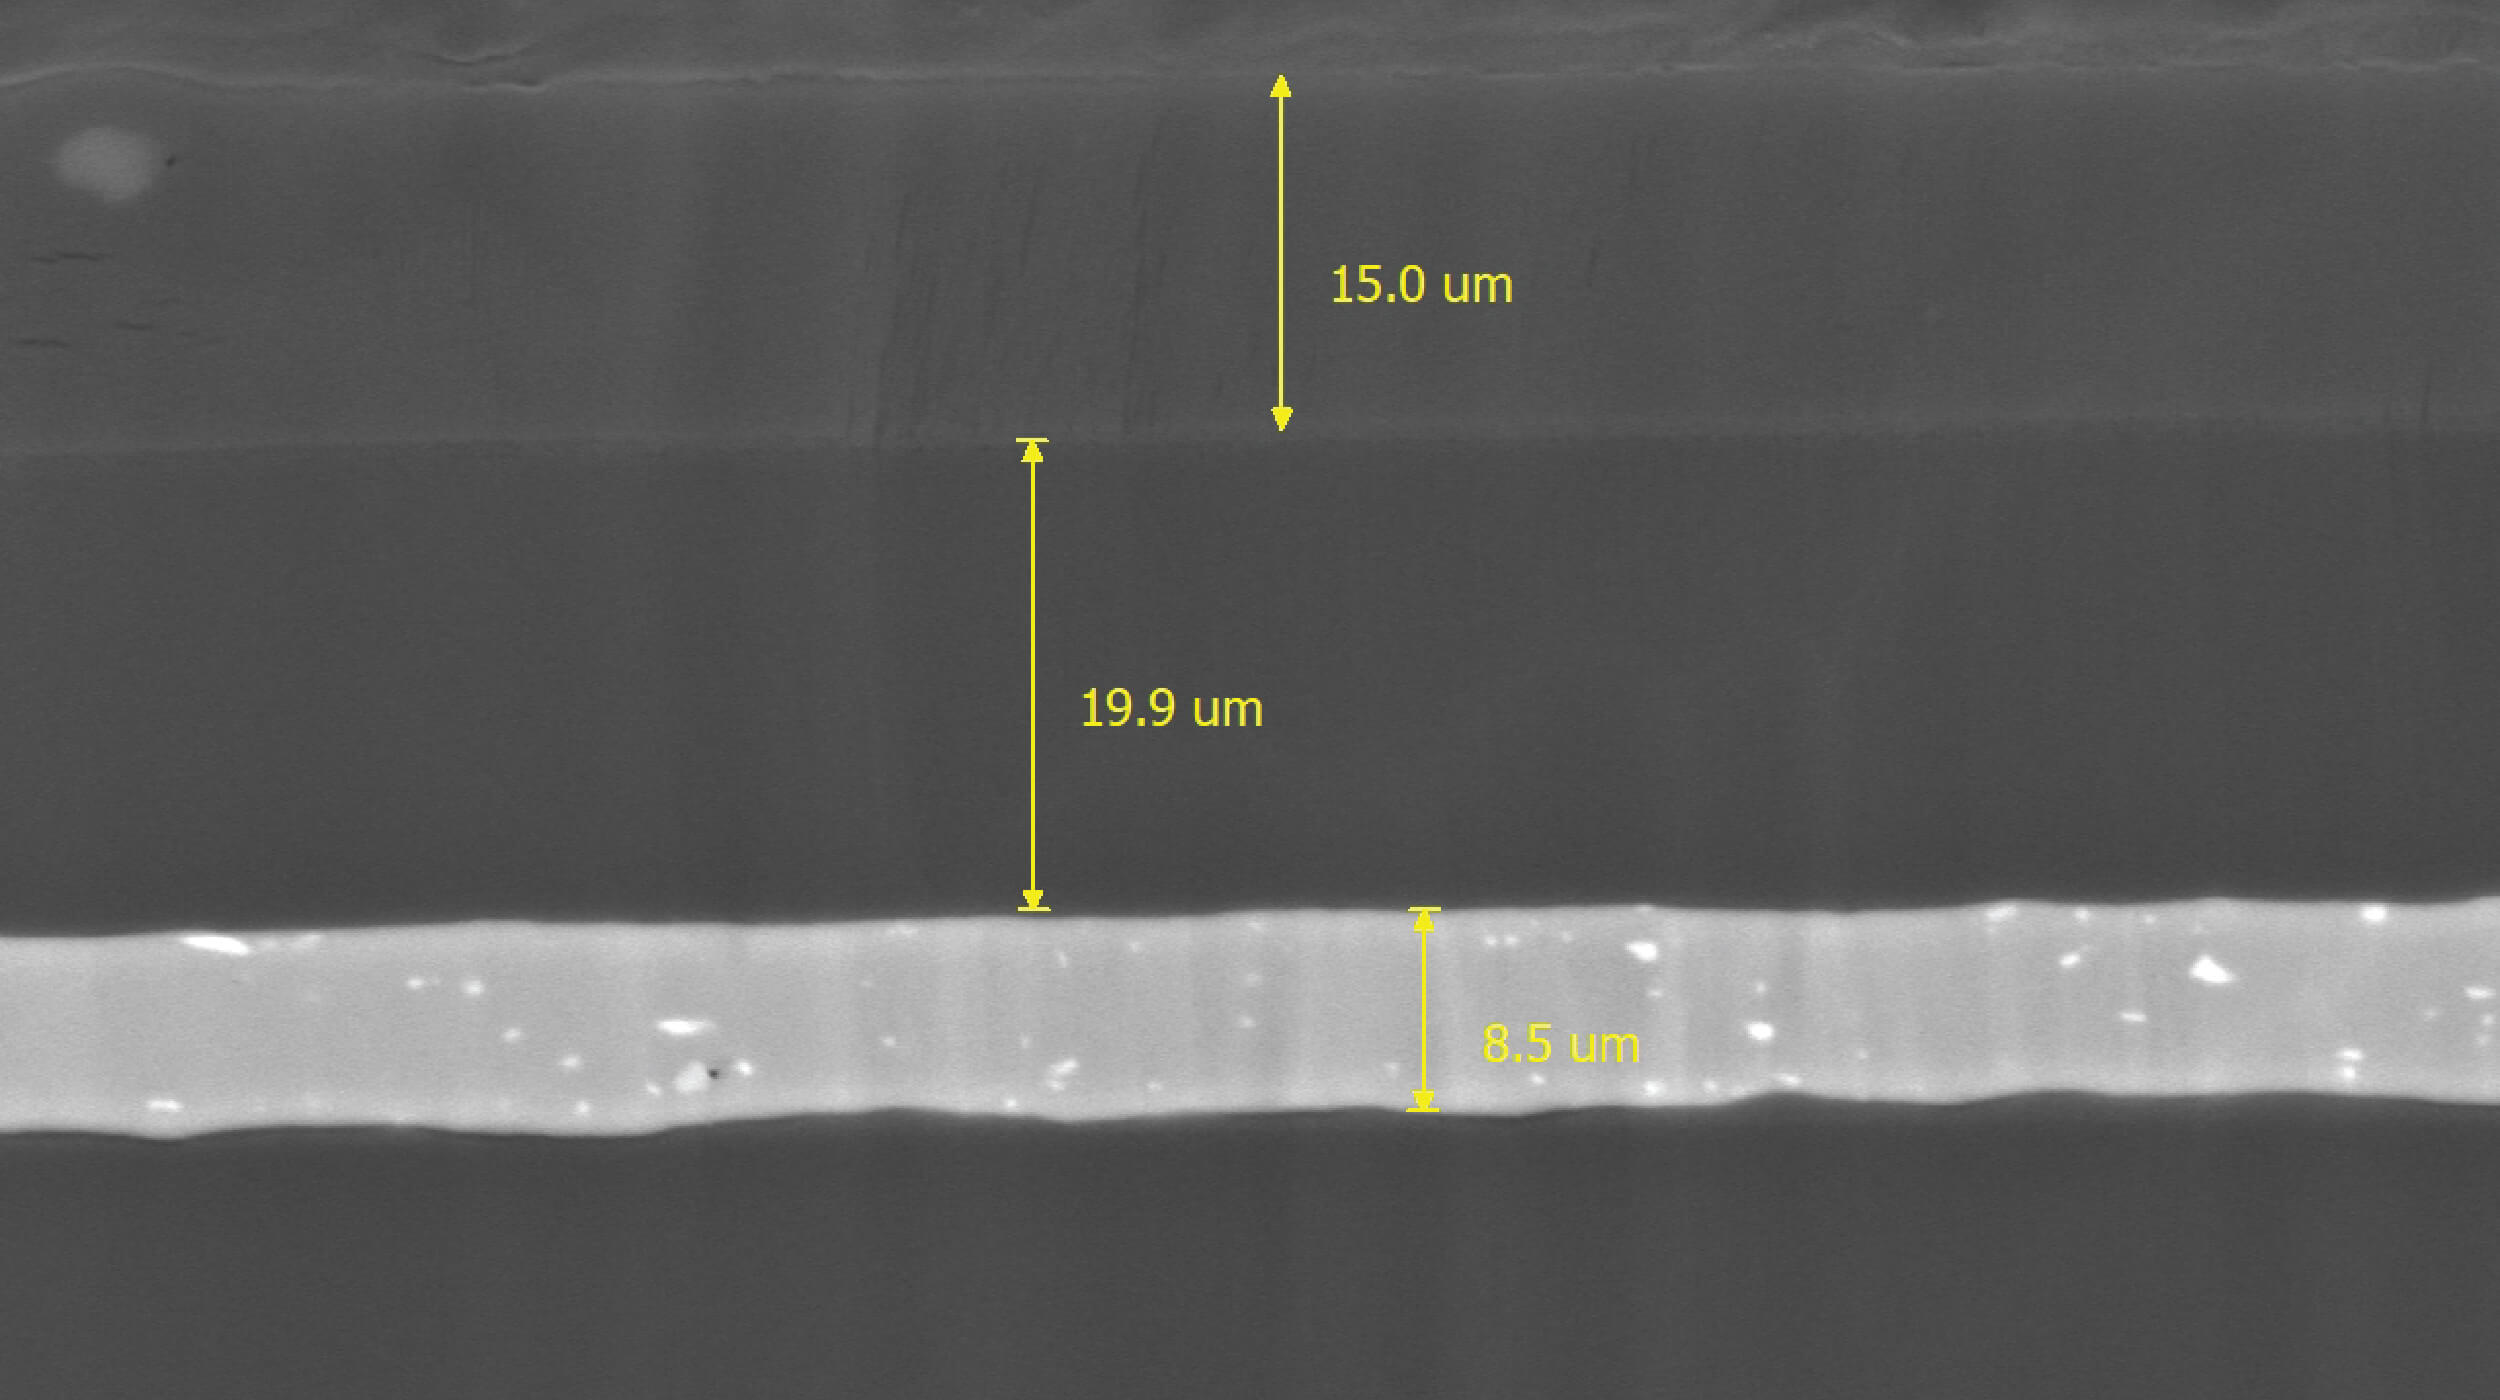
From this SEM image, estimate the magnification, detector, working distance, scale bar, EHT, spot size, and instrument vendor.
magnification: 1.9 K X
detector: BSE-COMPO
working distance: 8.9 mm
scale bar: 10000 nm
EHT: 20 kV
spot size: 12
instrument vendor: other